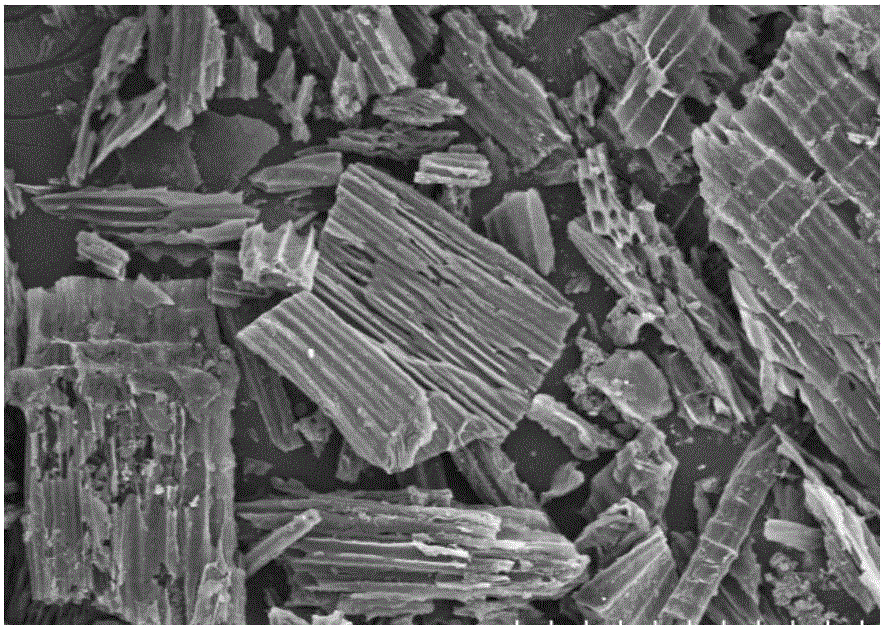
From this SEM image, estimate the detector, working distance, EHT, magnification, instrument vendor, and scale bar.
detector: SE(M,LA0)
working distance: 13.9 mm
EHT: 10 kV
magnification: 0.25 K X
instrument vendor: Hitachi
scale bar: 200000 nm